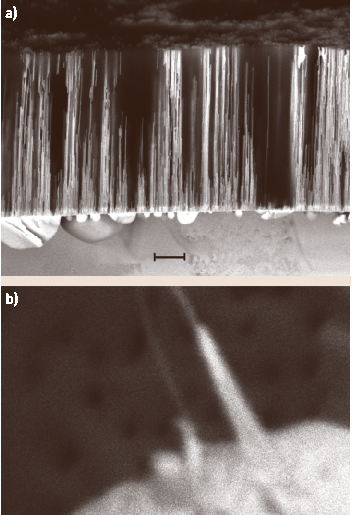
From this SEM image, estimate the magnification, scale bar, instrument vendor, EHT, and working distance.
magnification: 50 K X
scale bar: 100 nm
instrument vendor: JEOL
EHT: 5 kV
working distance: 8 mm